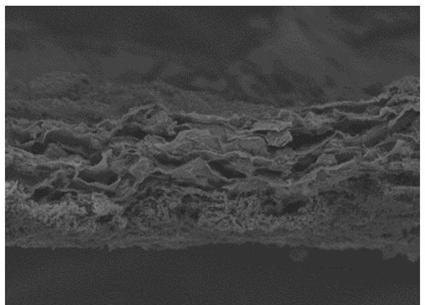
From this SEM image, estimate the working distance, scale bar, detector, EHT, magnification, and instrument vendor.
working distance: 7.6 mm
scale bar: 50000 nm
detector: SE(M)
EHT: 5 kV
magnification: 1 K X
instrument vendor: Hitachi